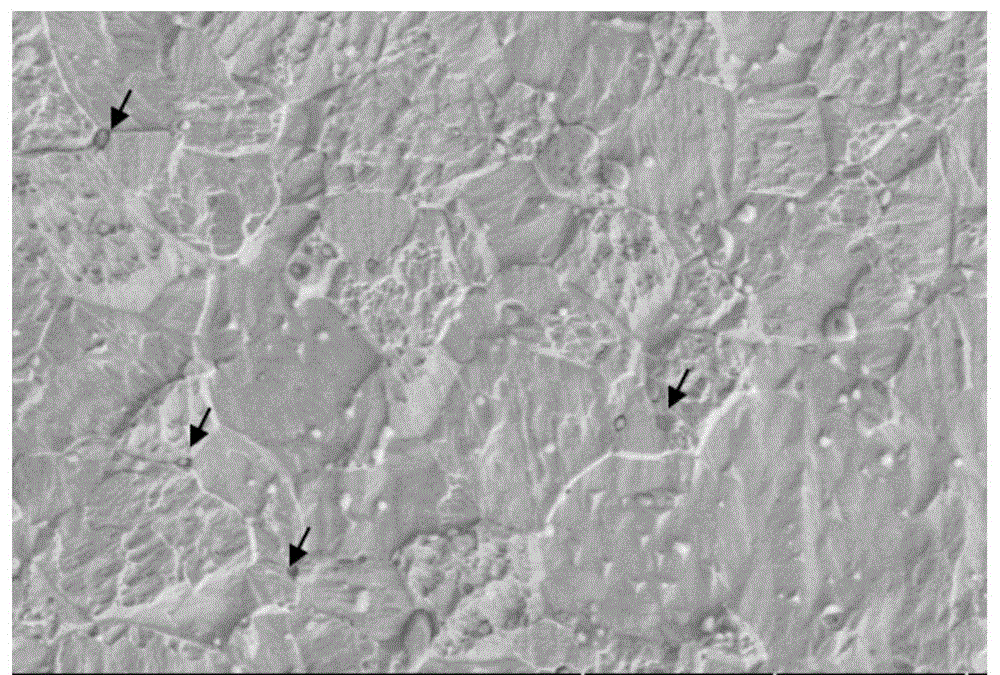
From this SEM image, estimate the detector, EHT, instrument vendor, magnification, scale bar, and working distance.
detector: SE(L)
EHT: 5 kV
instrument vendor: Hitachi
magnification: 10 K X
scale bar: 5000 nm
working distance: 11.4 mm